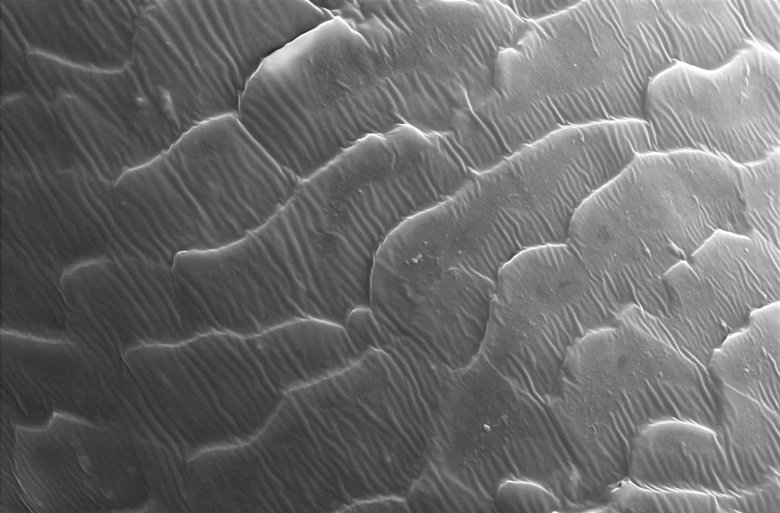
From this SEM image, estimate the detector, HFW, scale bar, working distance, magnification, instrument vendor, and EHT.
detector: ETD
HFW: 63.5 µm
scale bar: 10000 nm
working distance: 8.1 mm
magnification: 2 K X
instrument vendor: Thermo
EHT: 15 kV